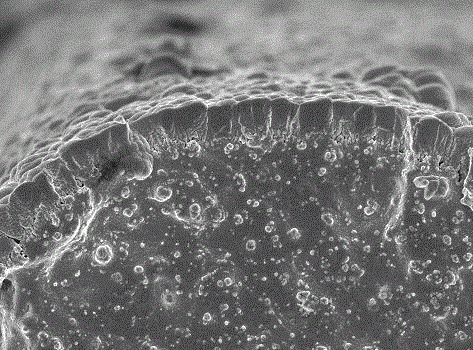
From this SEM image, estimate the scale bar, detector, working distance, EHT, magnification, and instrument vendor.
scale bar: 1000 nm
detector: SEI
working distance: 8 mm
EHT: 5 kV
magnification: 10 K X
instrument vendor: JEOL